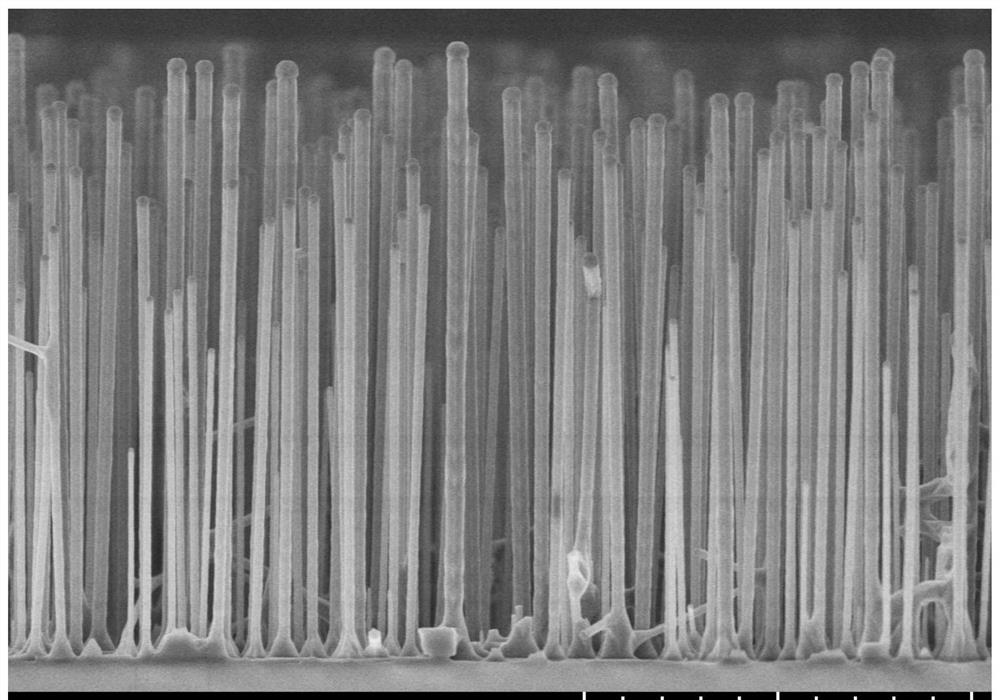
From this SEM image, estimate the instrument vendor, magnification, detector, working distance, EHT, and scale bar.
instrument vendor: Hitachi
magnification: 10 K X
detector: SE(UL)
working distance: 8.5 mm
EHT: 5 kV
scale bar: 5000 nm